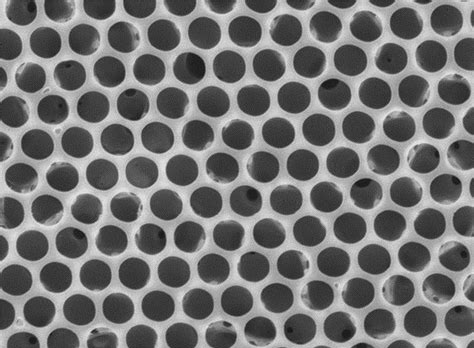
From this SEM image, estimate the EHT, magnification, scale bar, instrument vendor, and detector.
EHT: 15 kV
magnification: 3 K X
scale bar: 1000 nm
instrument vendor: JEOL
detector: SEI